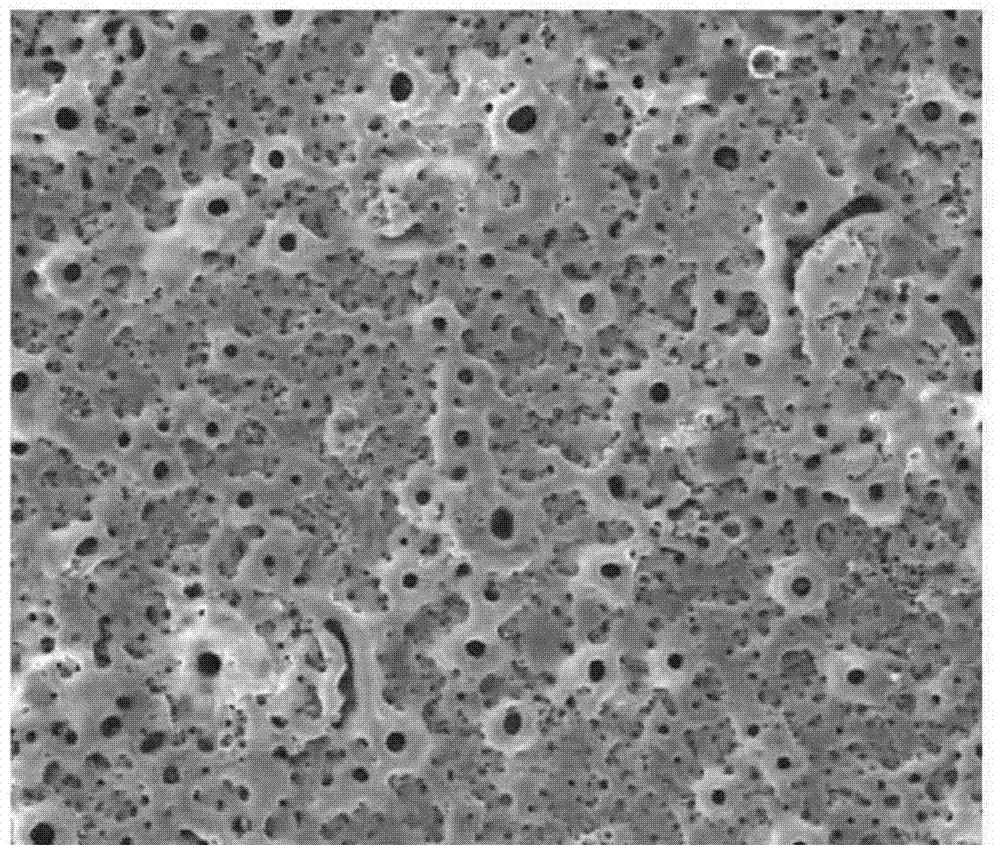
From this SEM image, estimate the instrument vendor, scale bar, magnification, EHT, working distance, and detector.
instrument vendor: FEI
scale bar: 50000 nm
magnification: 1 K X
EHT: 20 kV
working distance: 11 mm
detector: LFD